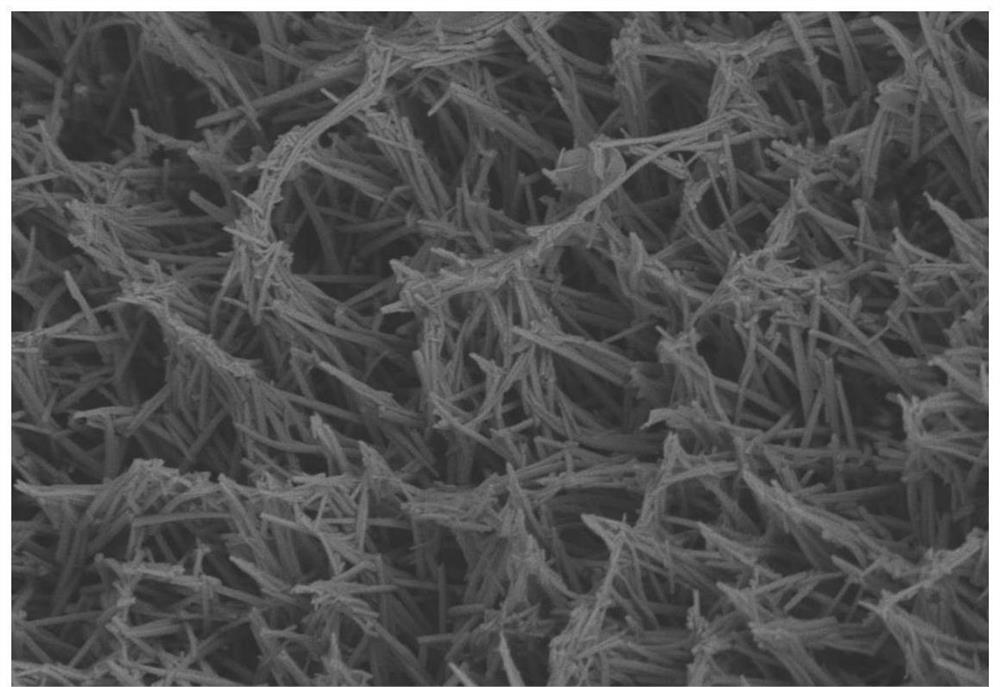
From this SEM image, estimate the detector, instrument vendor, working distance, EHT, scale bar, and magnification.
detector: SE2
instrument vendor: Zeiss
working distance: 9.4 mm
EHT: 3 kV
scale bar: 1000 nm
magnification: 10 K X